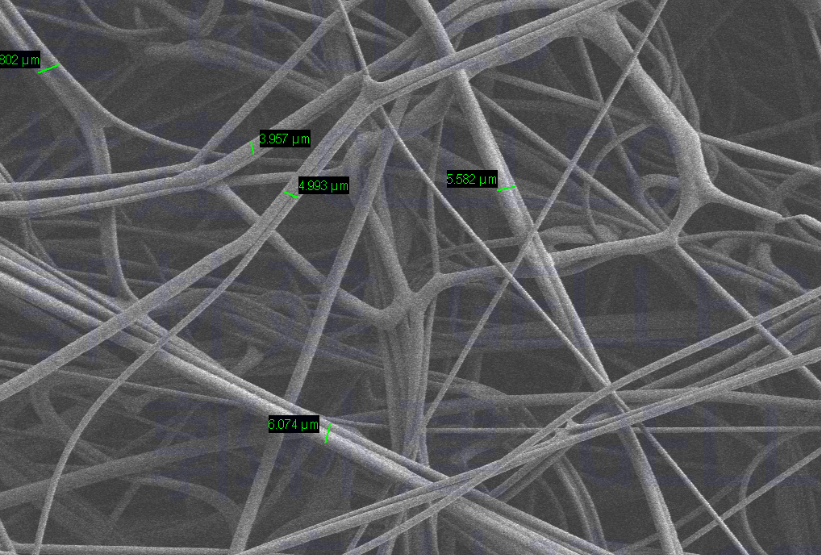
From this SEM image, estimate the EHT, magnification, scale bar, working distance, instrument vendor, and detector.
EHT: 1 kV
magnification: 0.5 K X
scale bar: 10000 nm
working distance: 4.5 mm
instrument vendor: Zeiss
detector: SE2